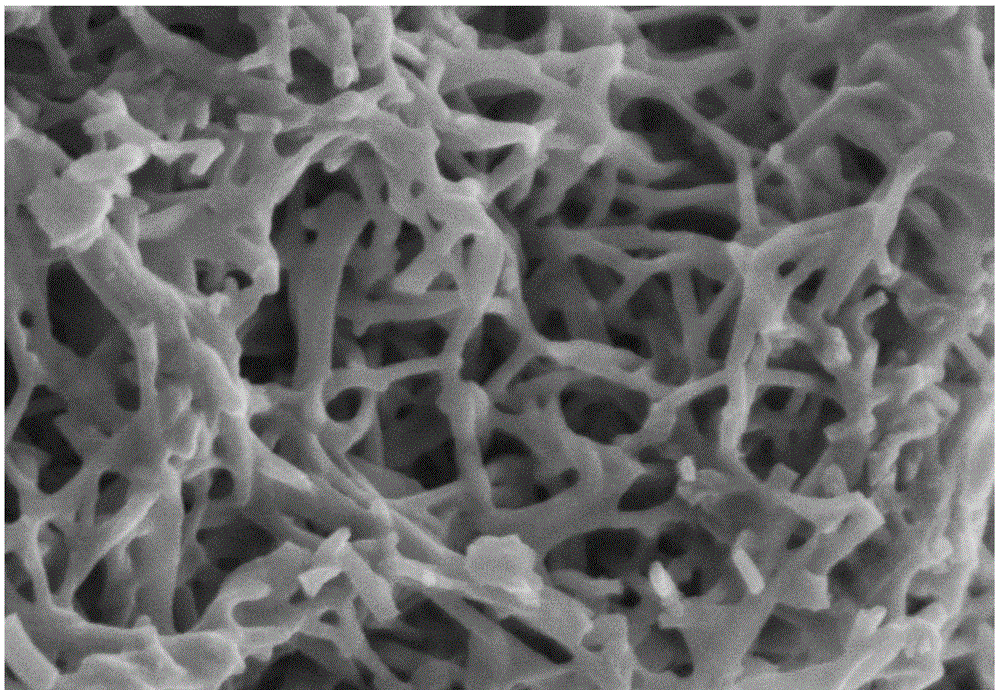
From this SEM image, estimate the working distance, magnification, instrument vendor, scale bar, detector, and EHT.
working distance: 5.5 mm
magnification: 100 K X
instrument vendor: Hitachi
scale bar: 500 nm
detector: SE+BSE(U)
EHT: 1.5 kV